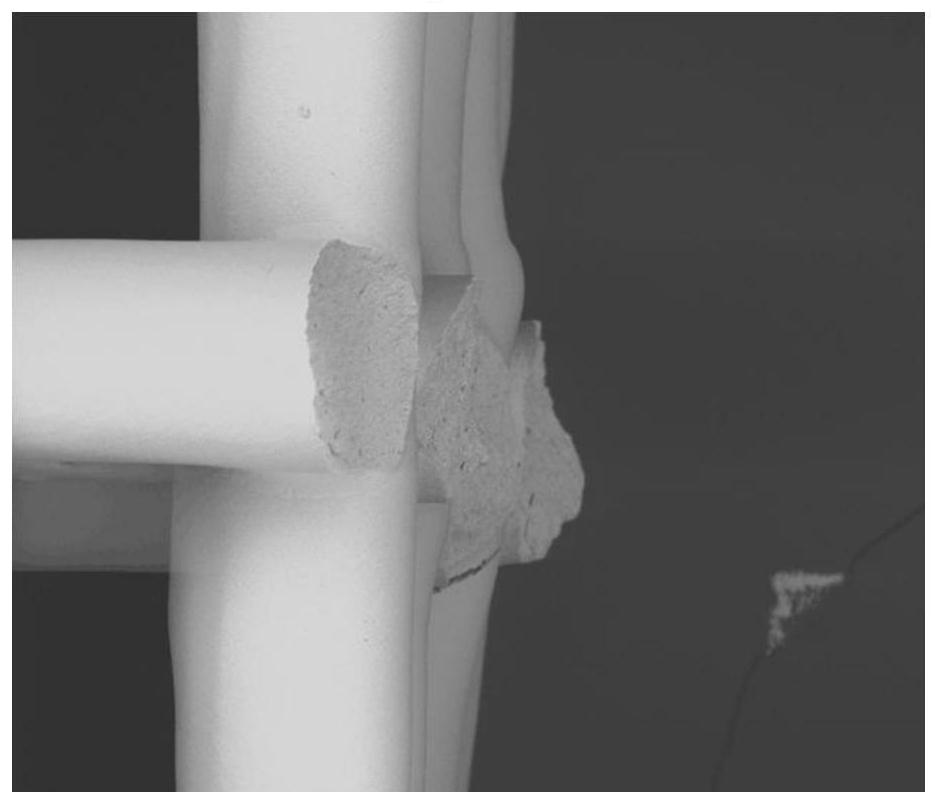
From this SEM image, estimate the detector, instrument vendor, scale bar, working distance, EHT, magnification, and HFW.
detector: BSED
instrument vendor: FEI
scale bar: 300000 nm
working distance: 5.6 mm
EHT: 18 kV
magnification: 0.25 K X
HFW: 960 µm